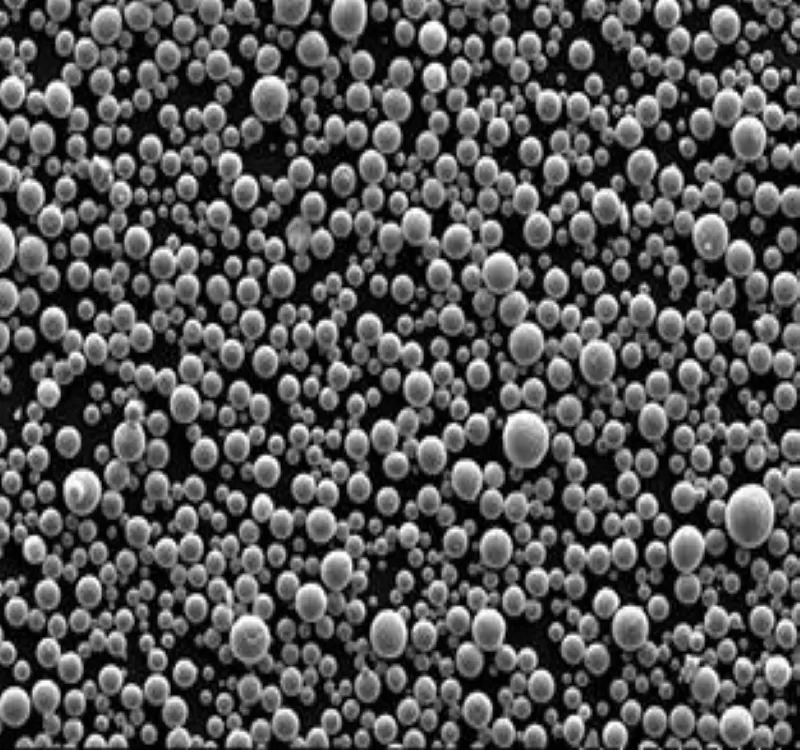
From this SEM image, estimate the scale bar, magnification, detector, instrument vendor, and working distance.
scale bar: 400000 nm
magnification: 0.2 K X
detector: LM(UL)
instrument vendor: Hitachi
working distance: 18.6 mm